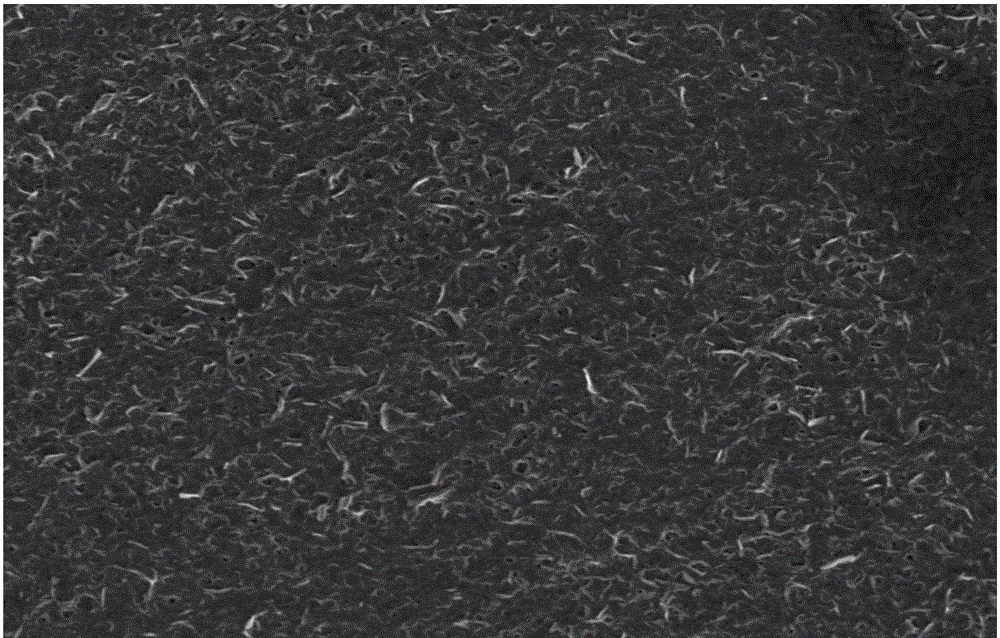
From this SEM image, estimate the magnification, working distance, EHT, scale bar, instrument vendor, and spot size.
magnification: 5 K X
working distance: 3.7 mm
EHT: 3 kV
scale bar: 20000 nm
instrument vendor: FEI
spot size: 3